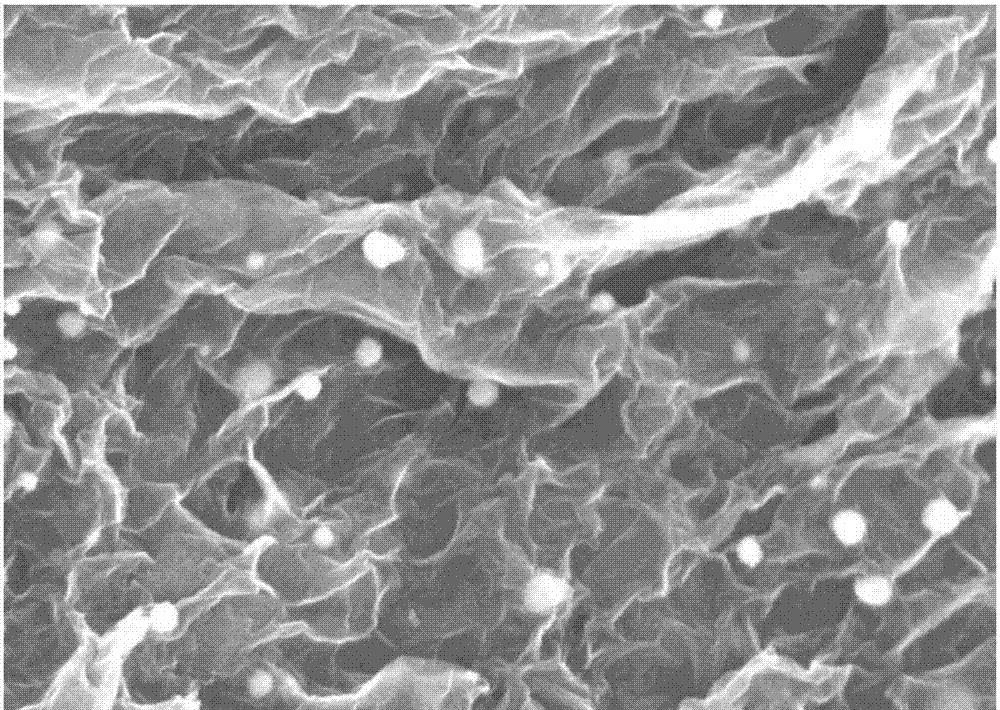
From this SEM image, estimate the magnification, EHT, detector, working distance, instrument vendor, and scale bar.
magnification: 50 K X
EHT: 5 kV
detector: SE(M)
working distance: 6.9 mm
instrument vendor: Hitachi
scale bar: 1000 nm